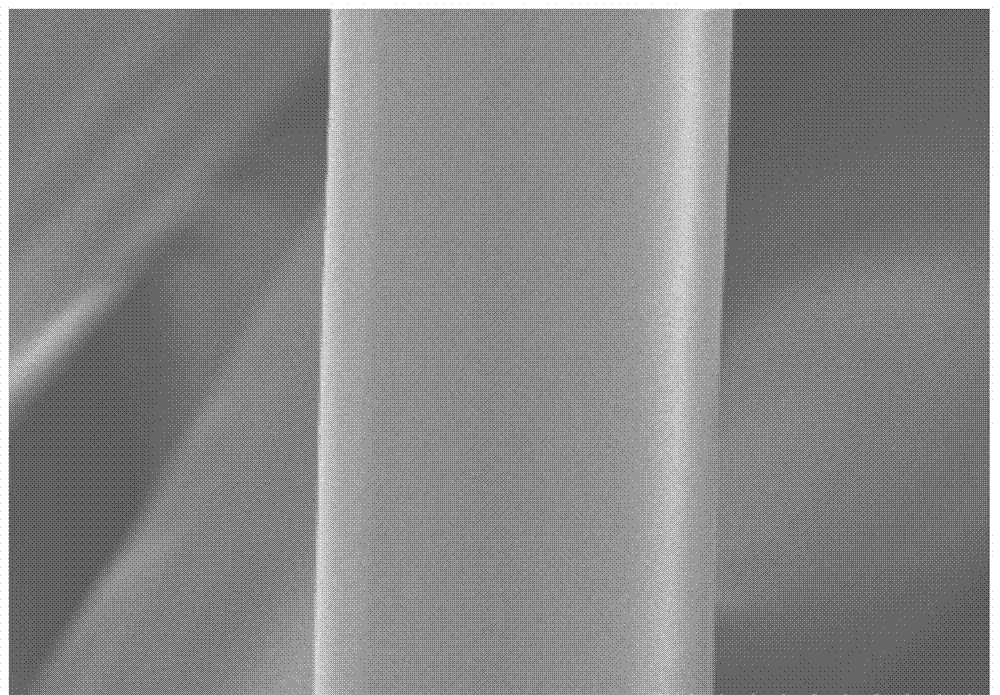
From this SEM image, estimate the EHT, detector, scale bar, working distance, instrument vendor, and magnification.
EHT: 5 kV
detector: SE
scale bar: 10000 nm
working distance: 10.4 mm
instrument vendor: Hitachi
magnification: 4 K X